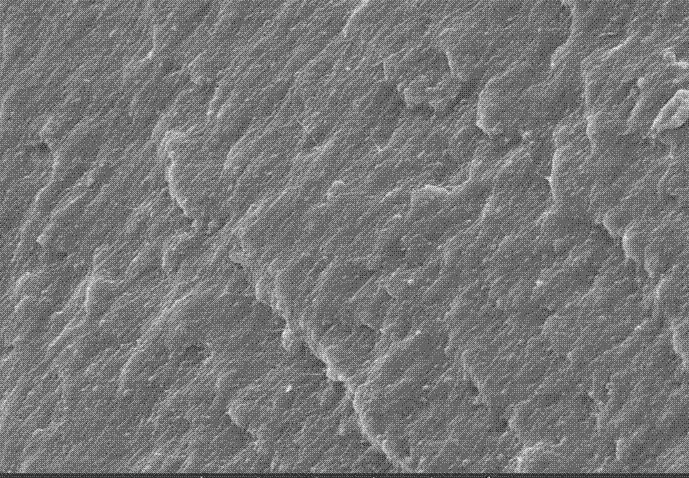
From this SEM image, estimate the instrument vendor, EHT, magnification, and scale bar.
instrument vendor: other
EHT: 20 kV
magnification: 0.5 K X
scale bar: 100000 nm